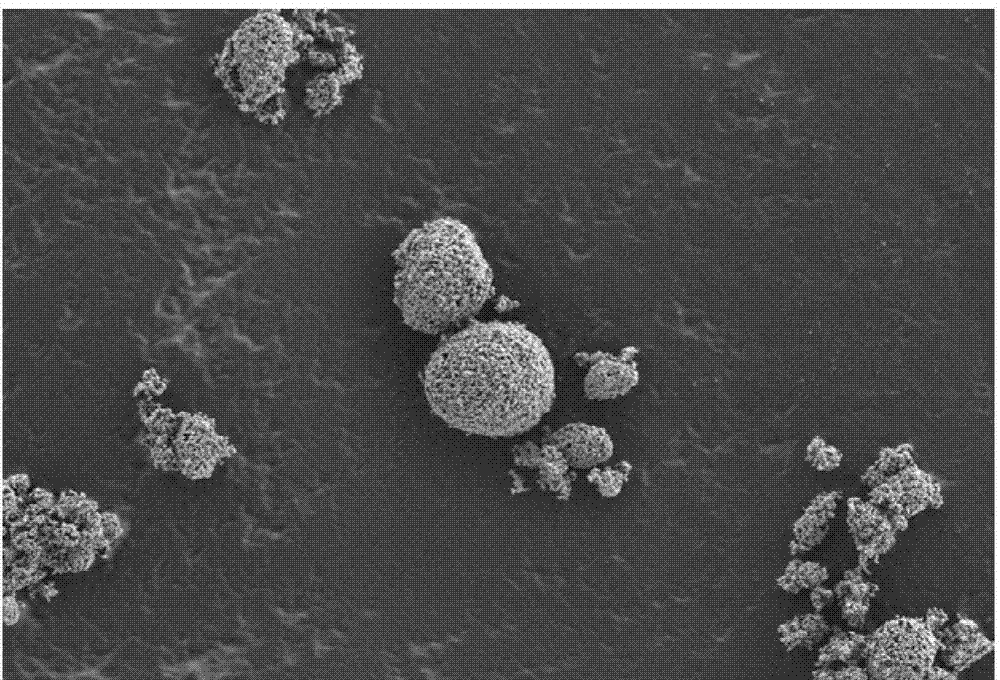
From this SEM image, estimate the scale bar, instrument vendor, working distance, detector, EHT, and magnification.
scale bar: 10000 nm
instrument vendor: Zeiss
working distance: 4.1 mm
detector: SE2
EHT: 3 kV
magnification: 1 K X